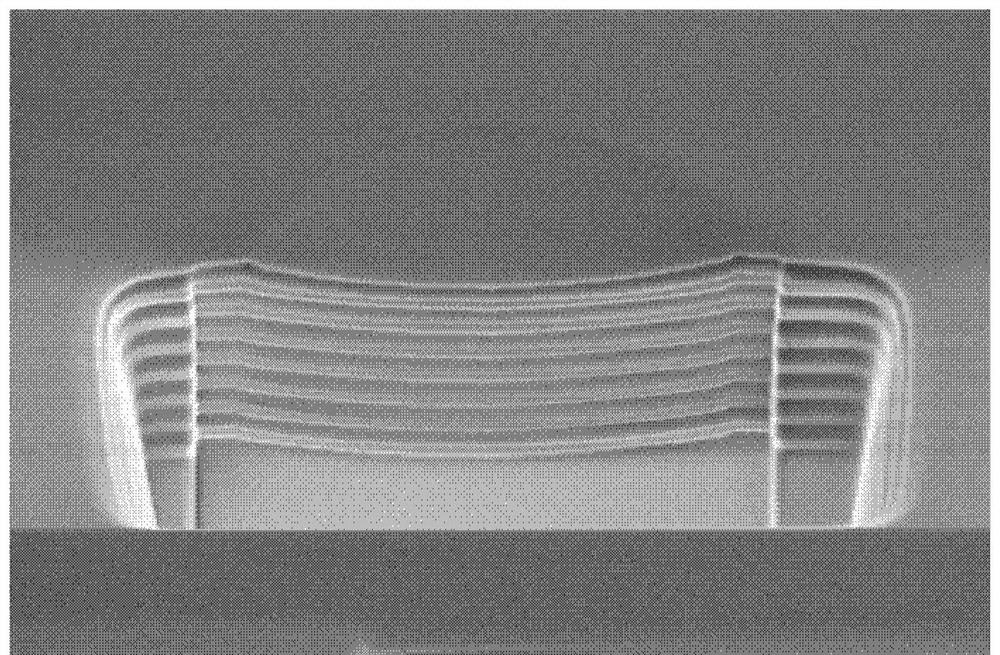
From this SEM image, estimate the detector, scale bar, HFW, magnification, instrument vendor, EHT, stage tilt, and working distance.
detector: TLD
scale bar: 1000 nm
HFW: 8.63 µm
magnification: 24 K X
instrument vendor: FEI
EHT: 2 kV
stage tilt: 52°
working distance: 4.1 mm